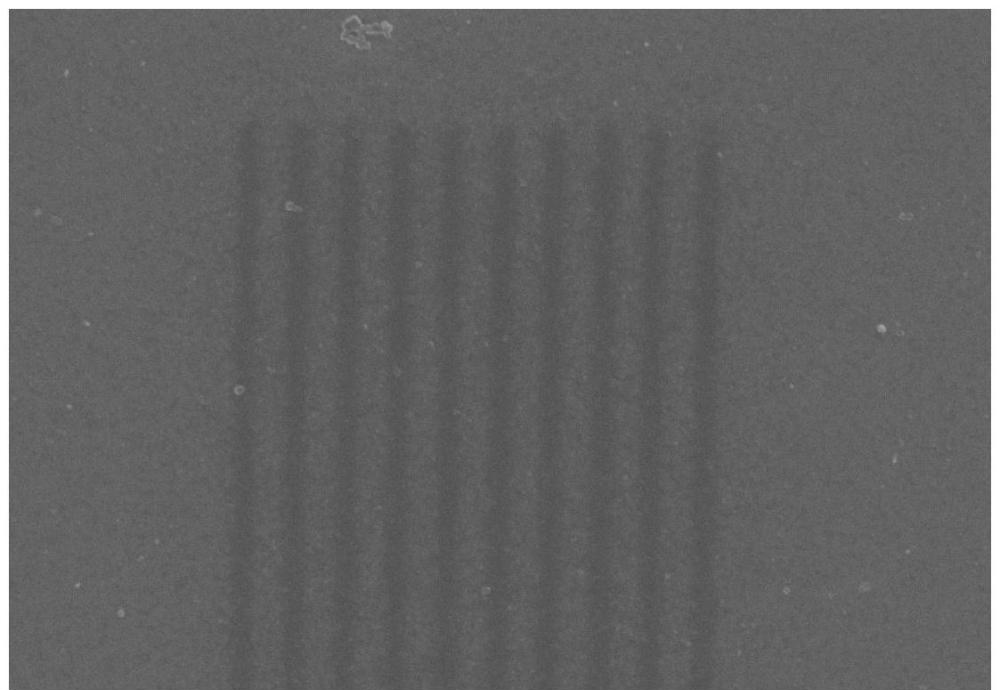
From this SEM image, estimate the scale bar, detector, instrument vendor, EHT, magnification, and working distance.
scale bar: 200 nm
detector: InLens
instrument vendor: Zeiss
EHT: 5 kV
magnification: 20 K X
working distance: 10.3 mm